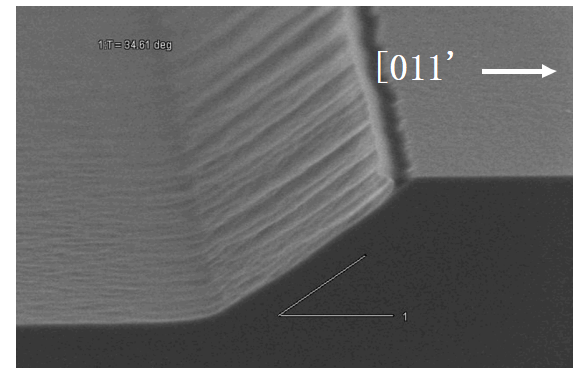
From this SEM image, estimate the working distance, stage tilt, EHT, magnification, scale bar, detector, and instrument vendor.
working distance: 6.2 mm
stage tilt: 34.61°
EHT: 2 kV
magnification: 45 K X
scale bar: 1000 nm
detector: SE(M)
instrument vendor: Hitachi